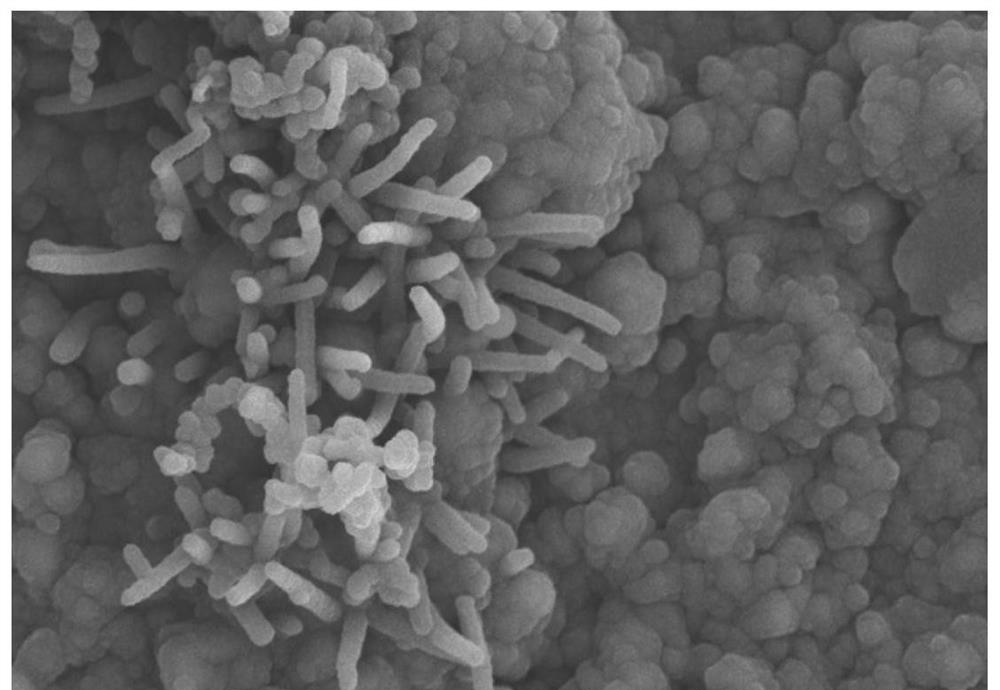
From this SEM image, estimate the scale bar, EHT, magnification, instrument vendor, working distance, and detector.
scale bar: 10000 nm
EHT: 15 kV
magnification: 5 K X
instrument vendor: Hitachi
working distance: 5.3 mm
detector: SE